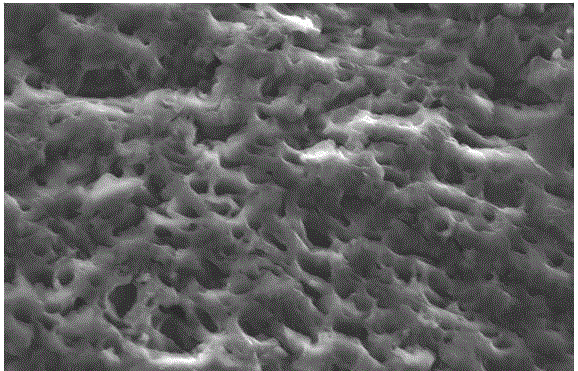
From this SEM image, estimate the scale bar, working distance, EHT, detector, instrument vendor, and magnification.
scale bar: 20000 nm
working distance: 9.4 mm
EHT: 20 kV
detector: SE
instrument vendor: FEI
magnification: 1 K X